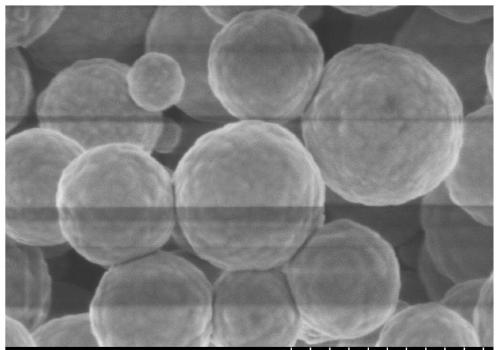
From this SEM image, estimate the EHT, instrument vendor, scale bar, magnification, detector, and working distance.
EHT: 1 kV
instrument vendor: Hitachi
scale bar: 1000 nm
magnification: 50 K X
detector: SE(M)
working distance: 3.2 mm